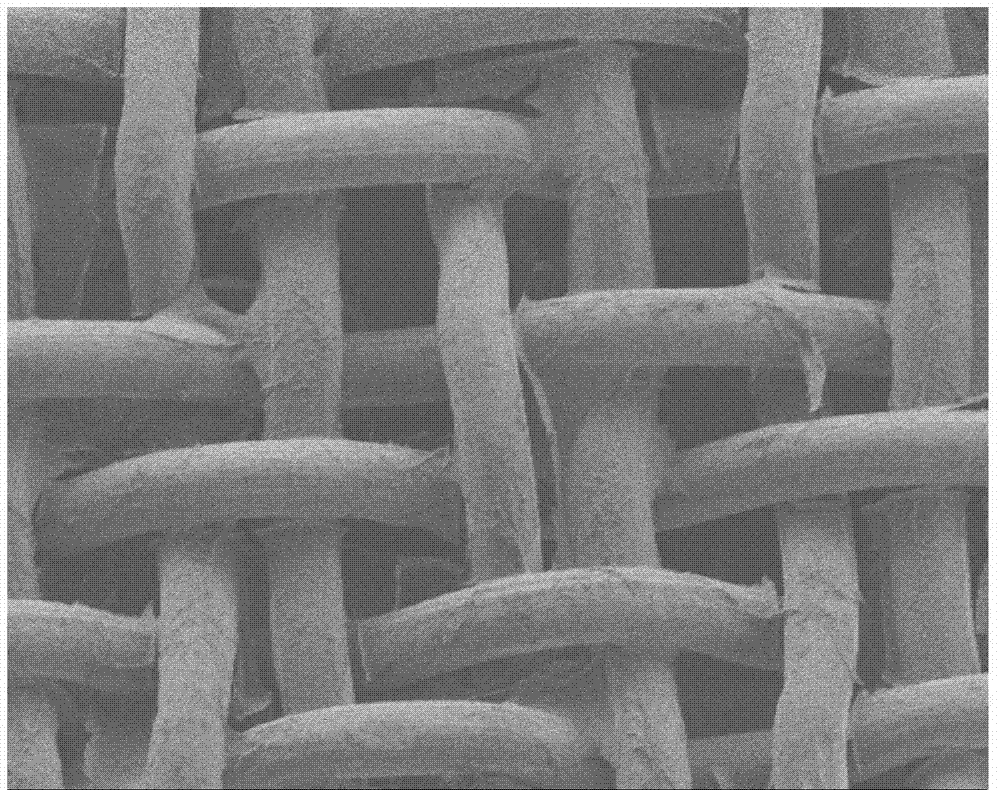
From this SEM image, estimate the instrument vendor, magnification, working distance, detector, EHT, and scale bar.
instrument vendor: JEOL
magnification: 0.22 K X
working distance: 6.1 mm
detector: LEI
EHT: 3 kV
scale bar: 100000 nm